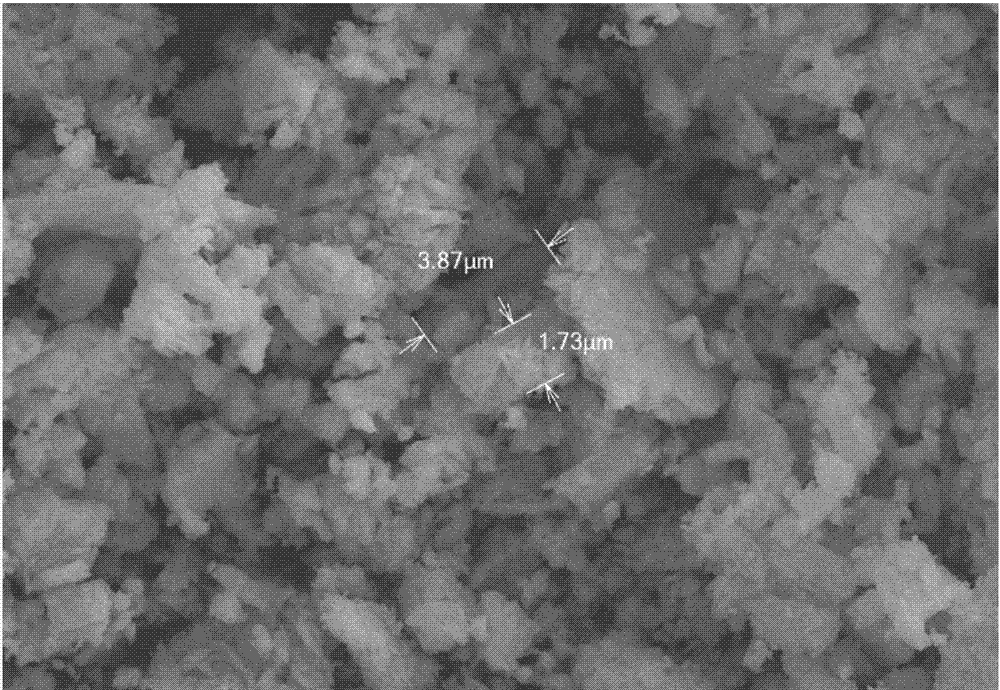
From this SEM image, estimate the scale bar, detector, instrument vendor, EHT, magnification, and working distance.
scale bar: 10000 nm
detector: SE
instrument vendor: Hitachi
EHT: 20 kV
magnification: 5 K X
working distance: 7.3 mm